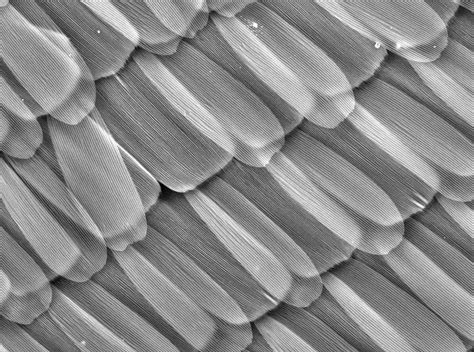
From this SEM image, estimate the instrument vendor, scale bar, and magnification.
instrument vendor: Hitachi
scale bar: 200000 nm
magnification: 0.4 K X